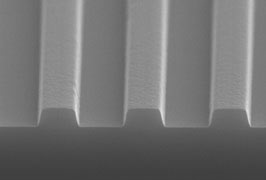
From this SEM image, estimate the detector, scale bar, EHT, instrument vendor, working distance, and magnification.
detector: S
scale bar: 1000 nm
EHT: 20 kV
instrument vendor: other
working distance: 7.4 mm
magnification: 10 K X